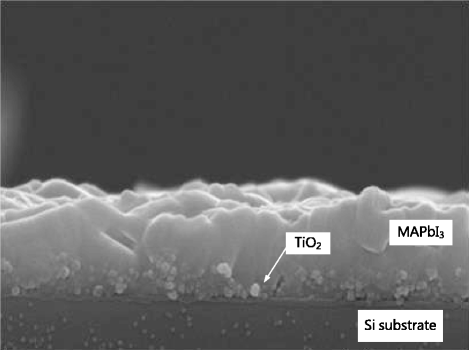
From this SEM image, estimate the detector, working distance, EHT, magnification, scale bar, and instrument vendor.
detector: SEI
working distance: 10 mm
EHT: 10 kV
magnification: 50 K X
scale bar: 100 nm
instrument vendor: JEOL